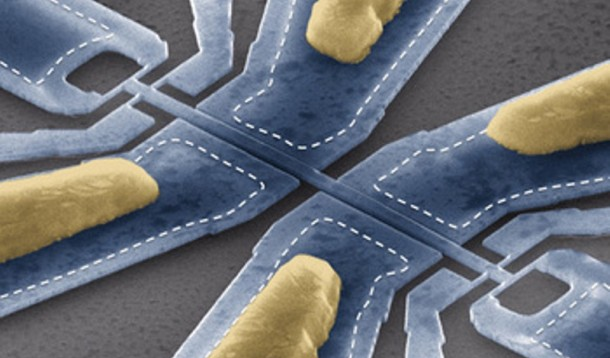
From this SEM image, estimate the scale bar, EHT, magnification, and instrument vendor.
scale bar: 2000 nm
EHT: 15 kV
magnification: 10 K X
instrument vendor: FEI